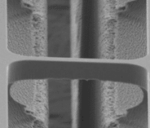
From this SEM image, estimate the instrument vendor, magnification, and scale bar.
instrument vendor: JEOL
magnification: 1 K X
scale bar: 10000 nm